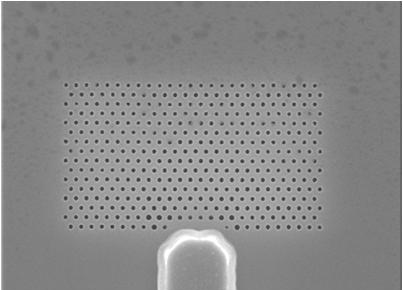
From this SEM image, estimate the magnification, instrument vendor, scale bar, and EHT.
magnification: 8 K X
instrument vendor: JEOL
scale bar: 3750 nm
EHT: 20 kV